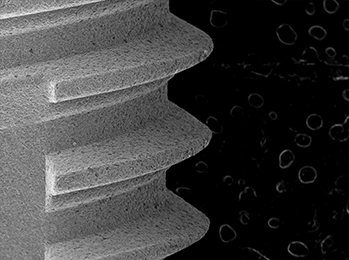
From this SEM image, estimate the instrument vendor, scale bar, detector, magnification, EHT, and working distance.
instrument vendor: other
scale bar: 1000000 nm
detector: SE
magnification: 0.055 K X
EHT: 15 kV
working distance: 41.6 mm